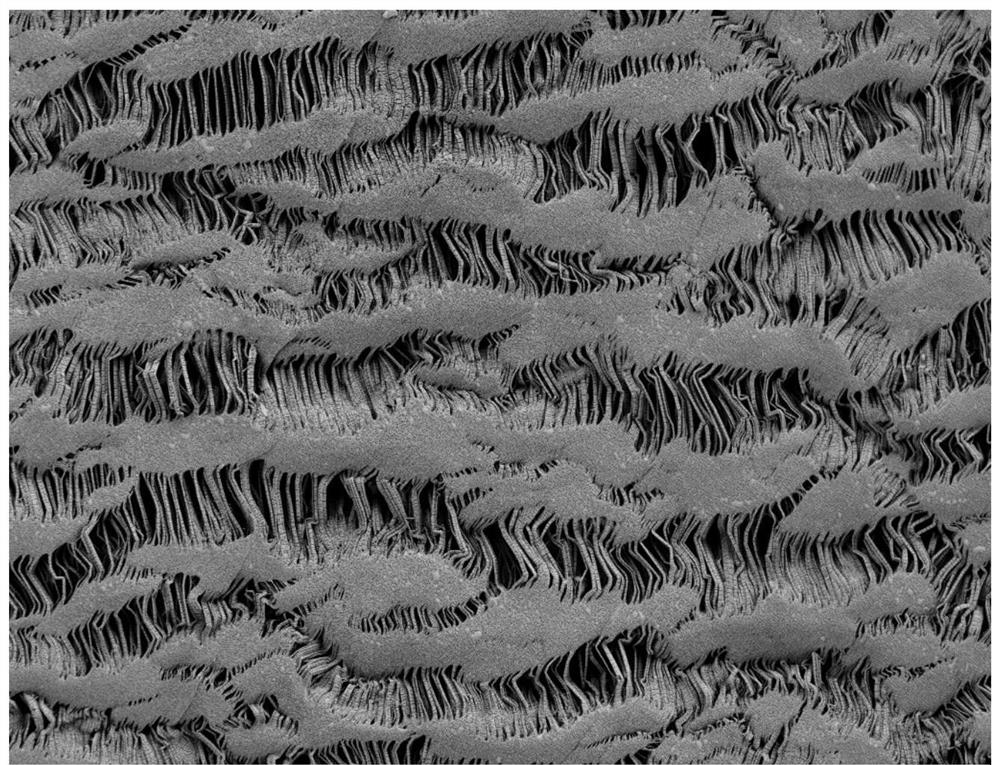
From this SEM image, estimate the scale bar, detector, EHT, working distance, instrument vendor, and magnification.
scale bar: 10000 nm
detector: SE2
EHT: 1 kV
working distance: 4.7 mm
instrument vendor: Zeiss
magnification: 1 K X